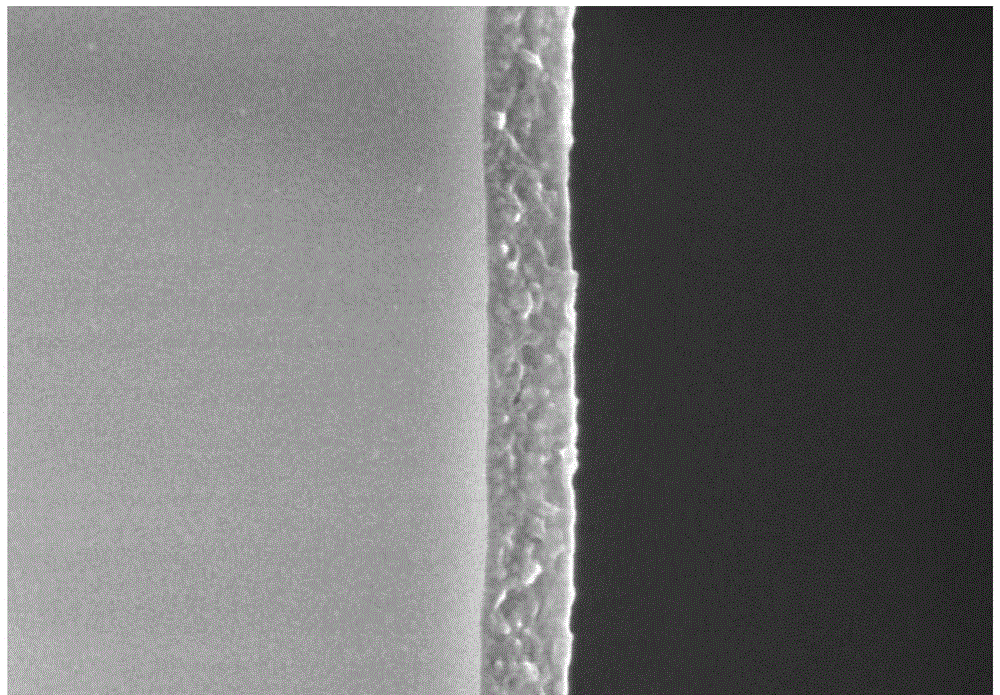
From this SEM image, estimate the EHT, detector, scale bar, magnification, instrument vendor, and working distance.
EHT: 3 kV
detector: SE(U)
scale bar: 500 nm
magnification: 100 K X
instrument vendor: Hitachi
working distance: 2.9 mm